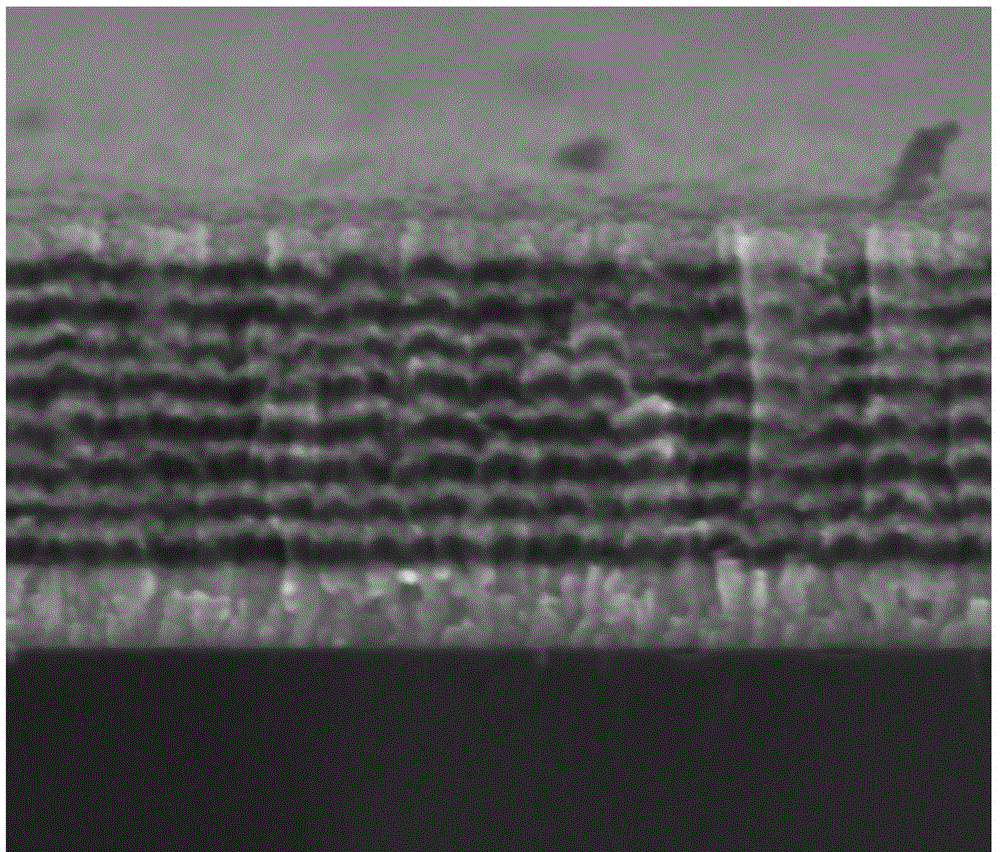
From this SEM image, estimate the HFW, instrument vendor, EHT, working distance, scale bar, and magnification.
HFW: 2.49 µm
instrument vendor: FEI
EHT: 5 kV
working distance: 5.5 mm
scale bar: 1000 nm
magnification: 120 K X